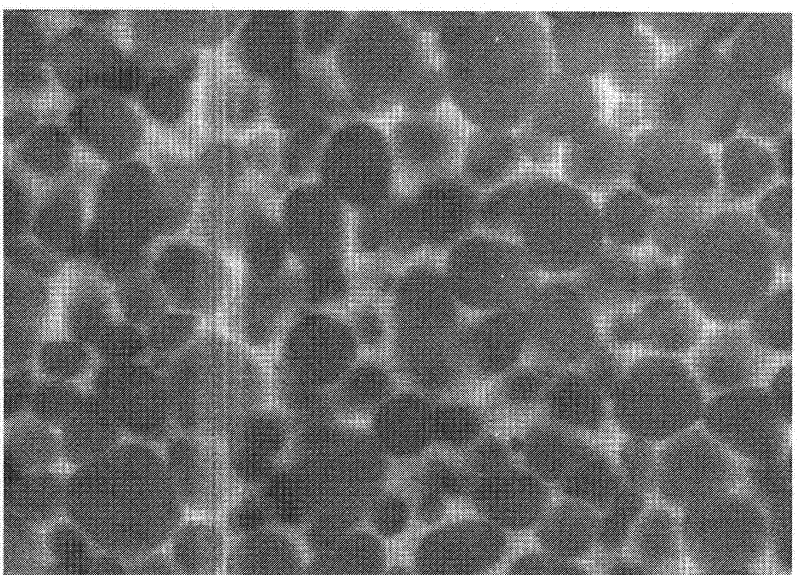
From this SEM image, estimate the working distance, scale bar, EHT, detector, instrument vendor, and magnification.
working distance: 17 mm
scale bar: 2000 nm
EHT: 15 kV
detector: SE(M)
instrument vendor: Hitachi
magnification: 20 K X